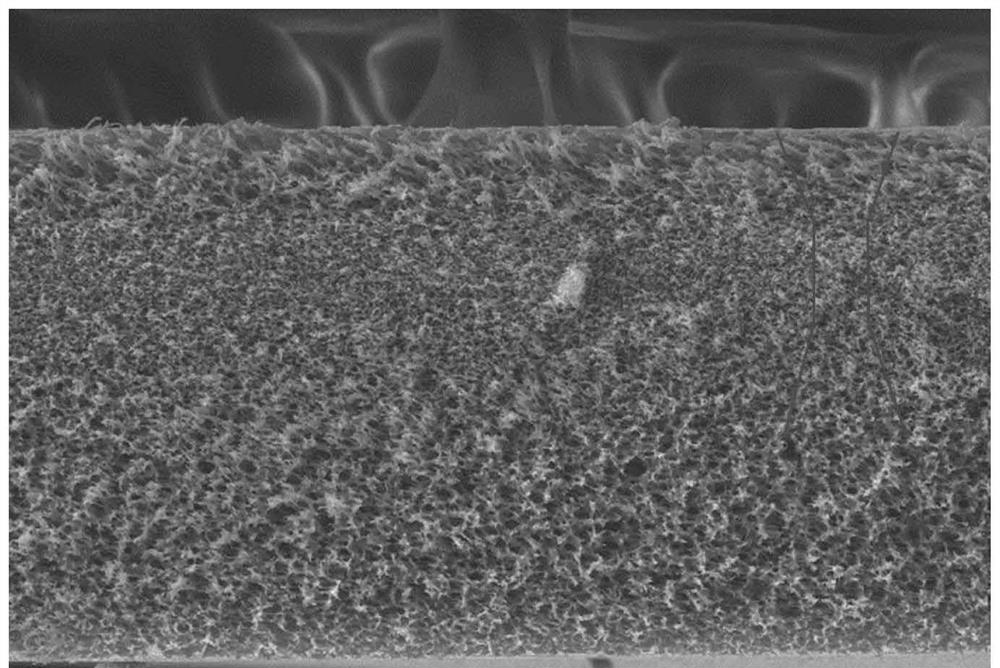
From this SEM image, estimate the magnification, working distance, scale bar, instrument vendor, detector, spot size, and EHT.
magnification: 0.596 K X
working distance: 10.5 mm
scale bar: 50000 nm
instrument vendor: FEI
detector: SE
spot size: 3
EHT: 15 kV